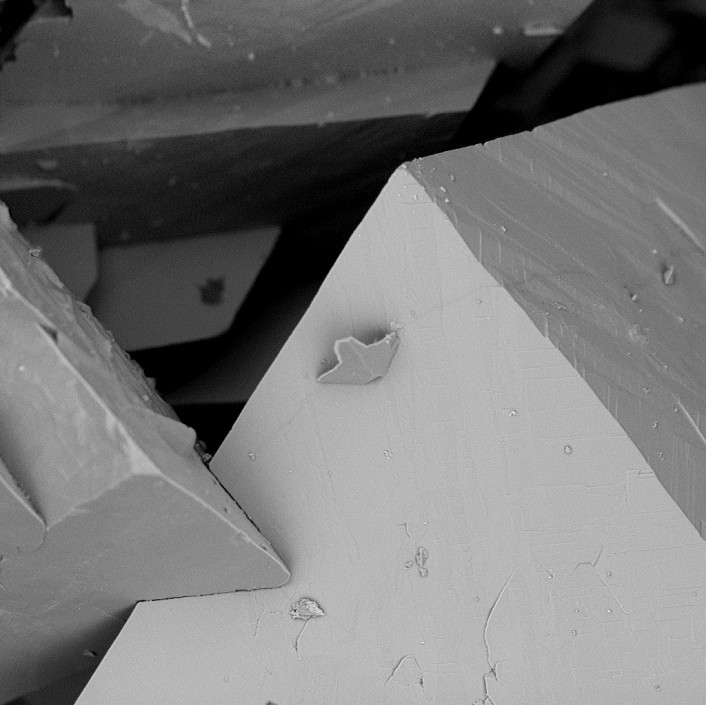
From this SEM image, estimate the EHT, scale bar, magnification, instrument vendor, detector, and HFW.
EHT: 15 kV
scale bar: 200000 nm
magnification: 0.38 K X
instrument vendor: other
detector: BSD Full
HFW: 720 µm